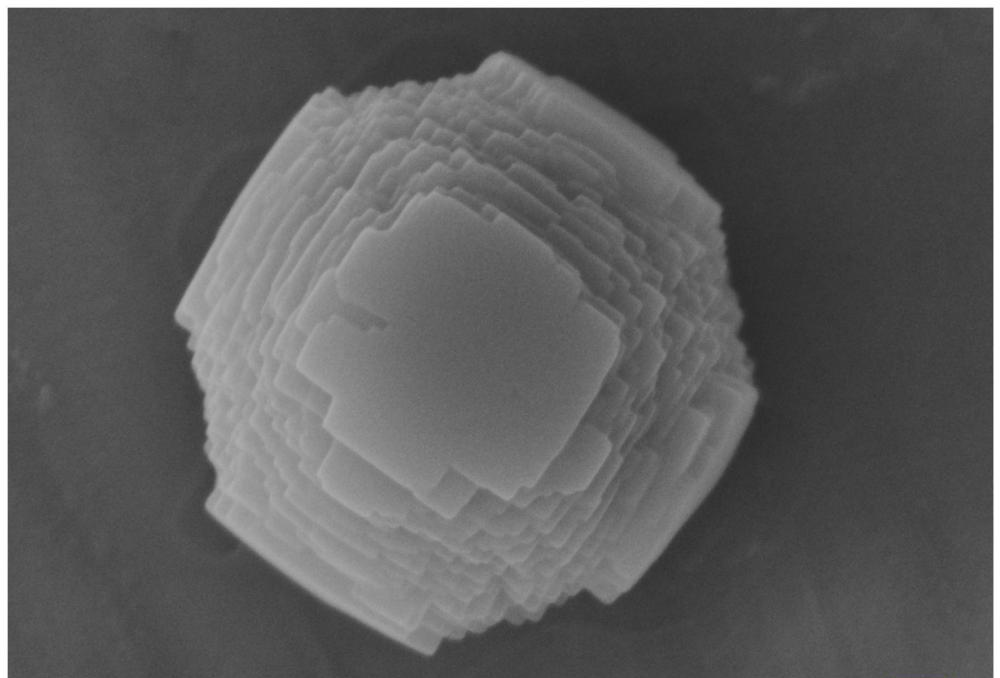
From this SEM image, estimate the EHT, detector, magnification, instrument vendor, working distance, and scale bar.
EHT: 5 kV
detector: InLens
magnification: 50 K X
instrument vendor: Zeiss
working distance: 7.4 mm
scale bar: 200 nm